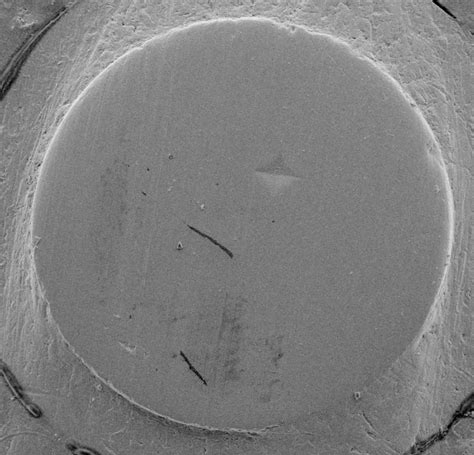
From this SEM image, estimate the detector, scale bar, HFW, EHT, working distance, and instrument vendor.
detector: SE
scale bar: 20000 nm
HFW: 144 µm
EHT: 5 kV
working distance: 15.06 mm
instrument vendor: Tescan